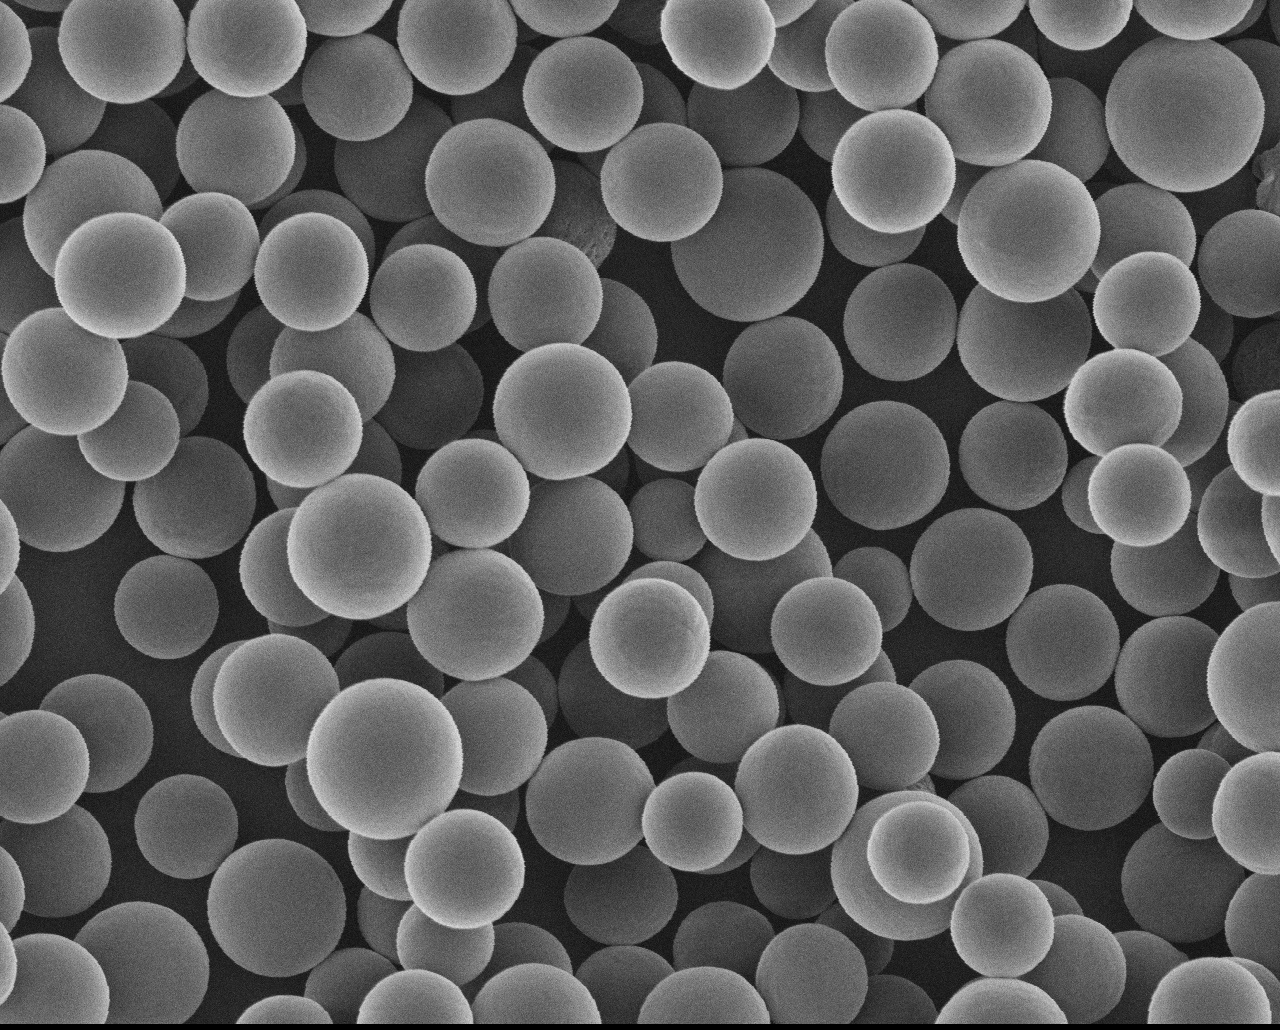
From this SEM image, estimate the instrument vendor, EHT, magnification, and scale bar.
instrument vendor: JEOL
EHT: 10 kV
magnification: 0.4 K X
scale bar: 50000 nm